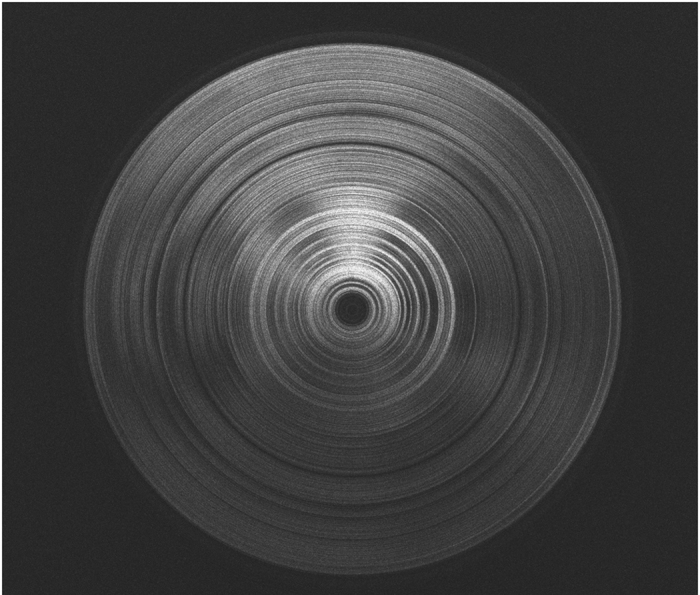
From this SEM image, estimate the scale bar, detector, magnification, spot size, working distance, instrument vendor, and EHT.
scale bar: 200000 nm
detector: ETD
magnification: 0.4 K X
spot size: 2.5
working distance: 4.5 mm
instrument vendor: FEI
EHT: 5 kV